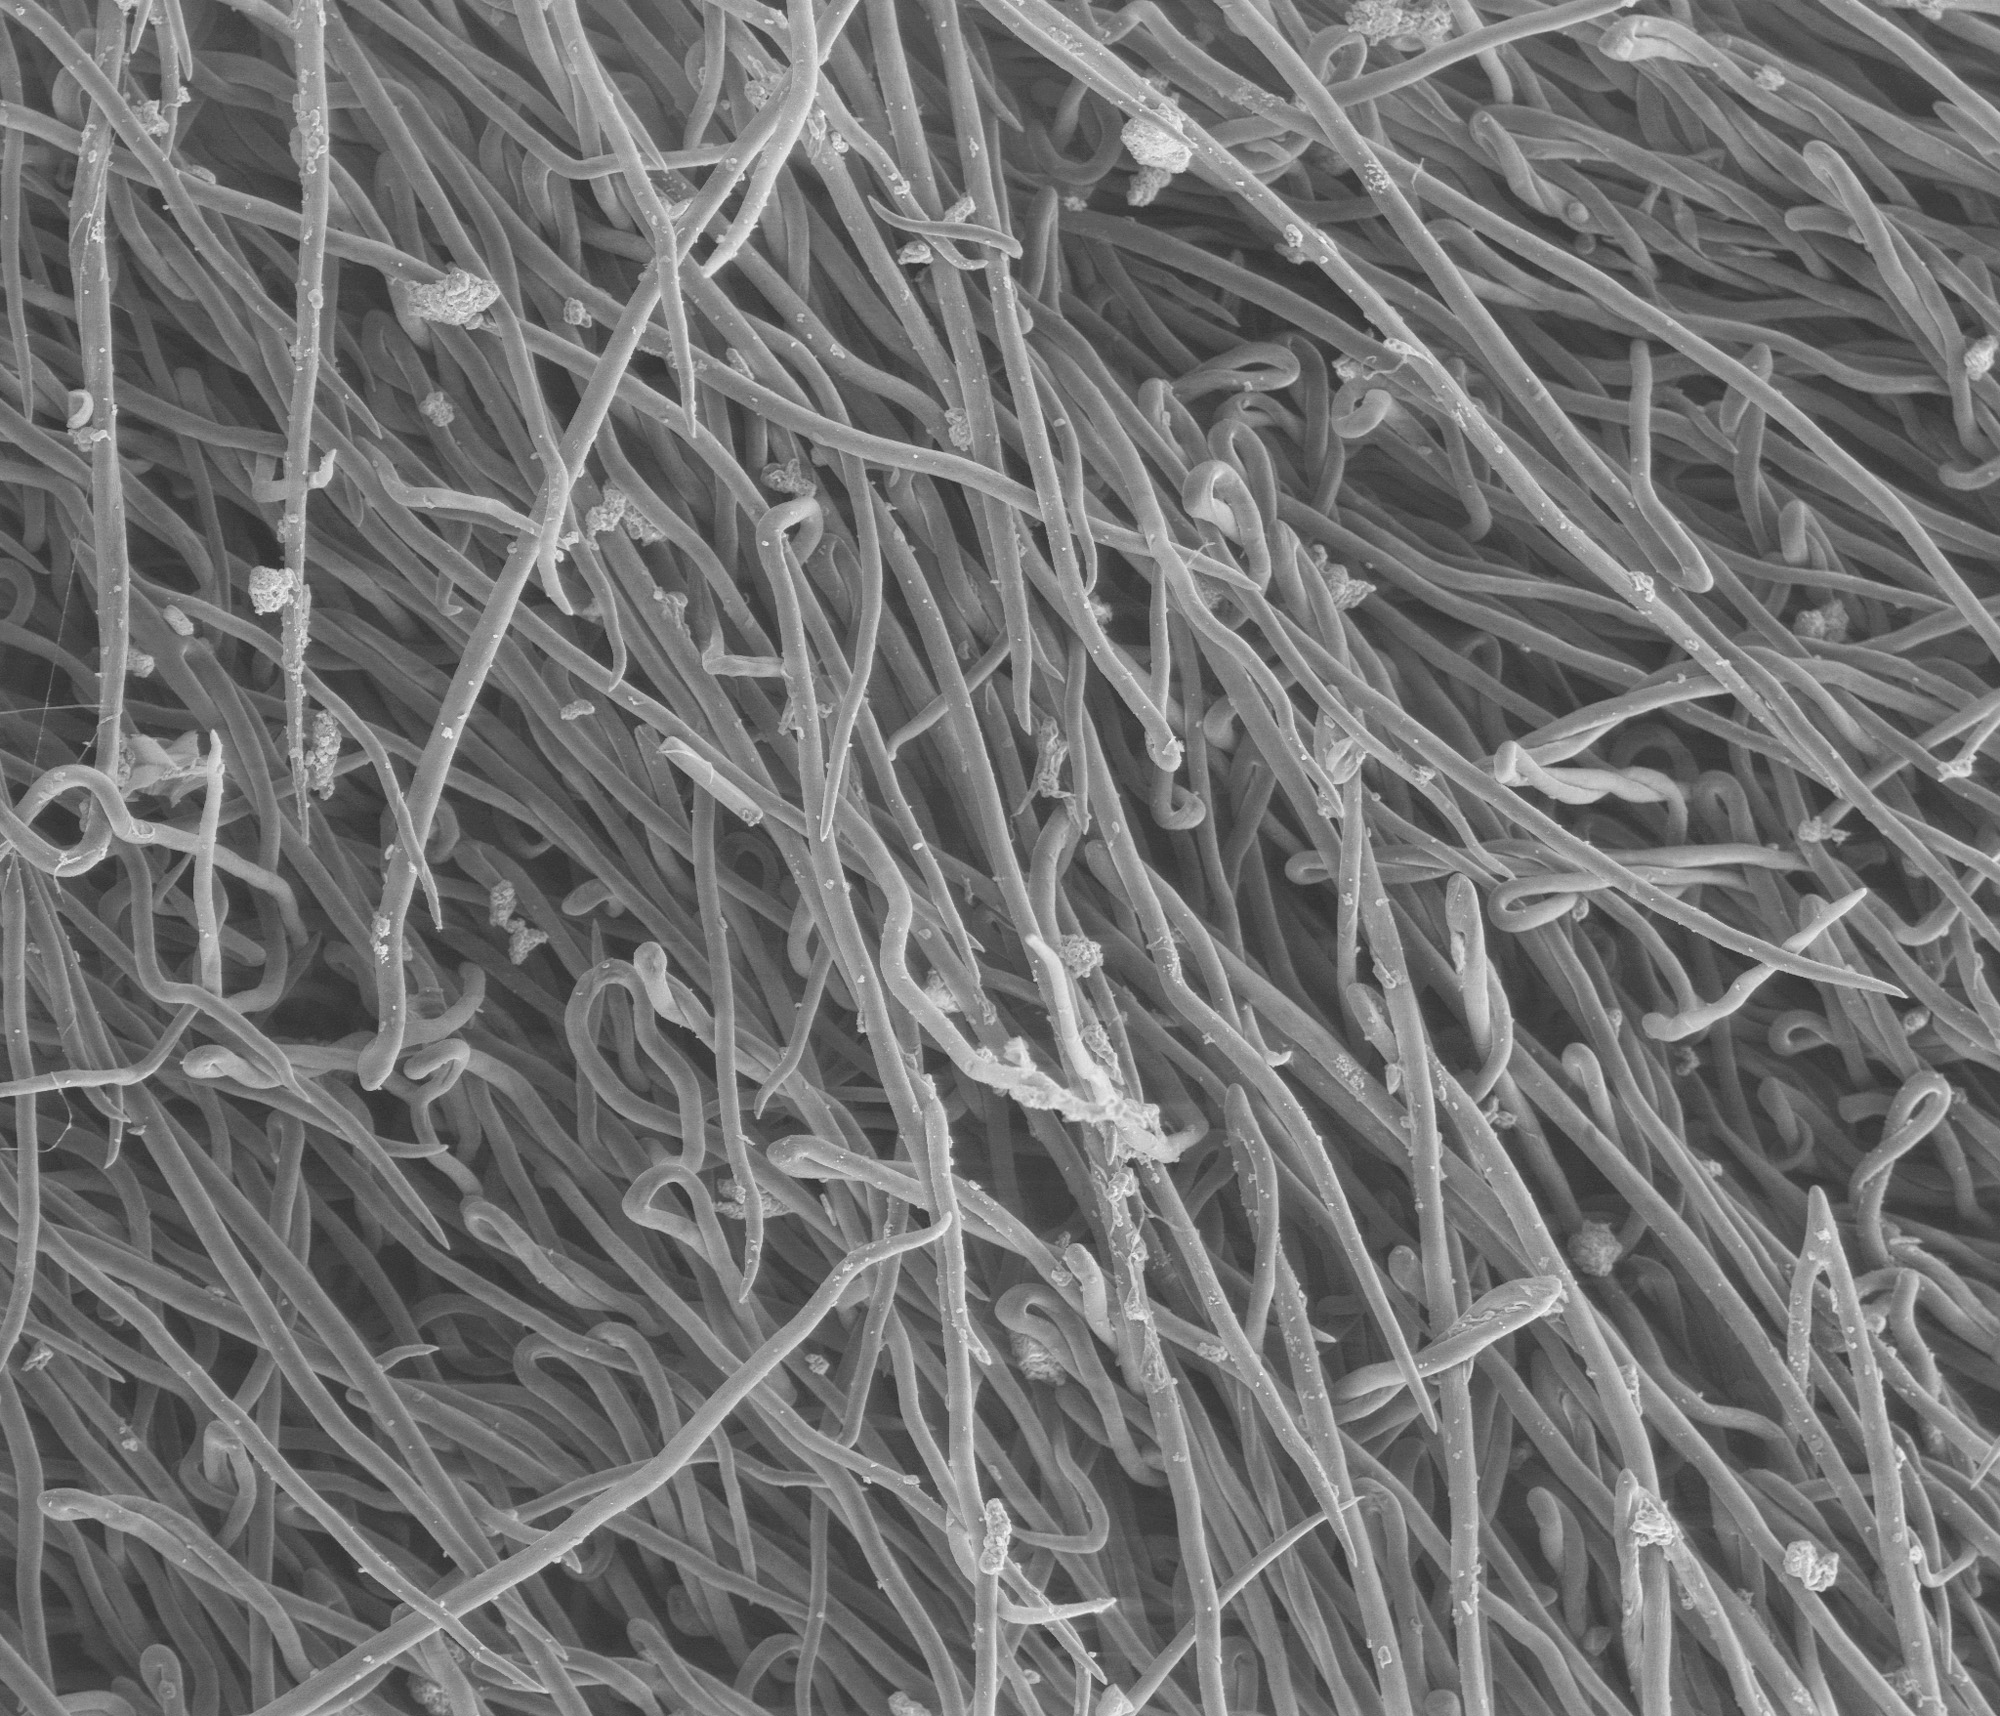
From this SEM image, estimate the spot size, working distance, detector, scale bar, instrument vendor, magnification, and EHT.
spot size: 4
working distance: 10.1 mm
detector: LFD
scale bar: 400000 nm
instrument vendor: FEI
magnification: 0.25 K X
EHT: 12.5 kV